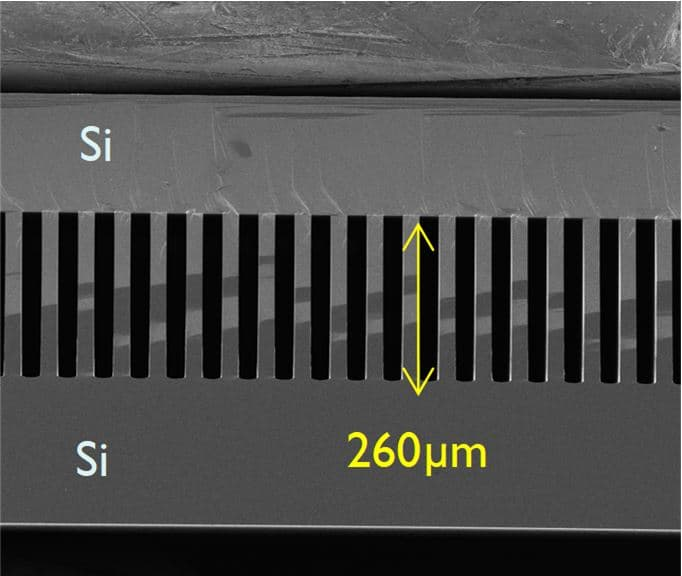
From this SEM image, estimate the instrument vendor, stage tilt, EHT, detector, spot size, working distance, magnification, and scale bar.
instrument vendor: FEI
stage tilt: -9°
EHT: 5 kV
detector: ETD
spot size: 3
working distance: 6.5 mm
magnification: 0.225 K X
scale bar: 400000 nm